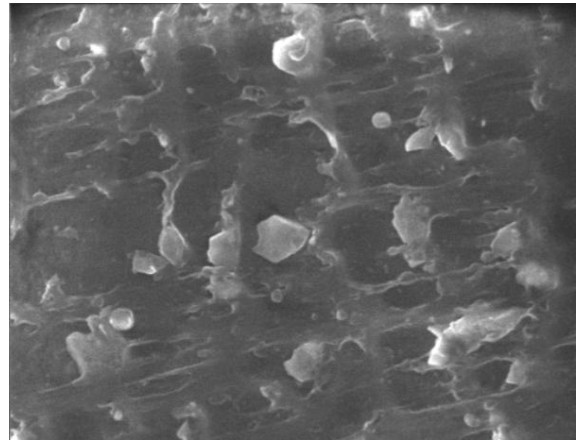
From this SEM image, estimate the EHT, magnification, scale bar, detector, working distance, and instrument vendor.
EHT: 15 kV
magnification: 8 K X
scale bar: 4000 nm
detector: SE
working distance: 10 mm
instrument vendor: Tescan